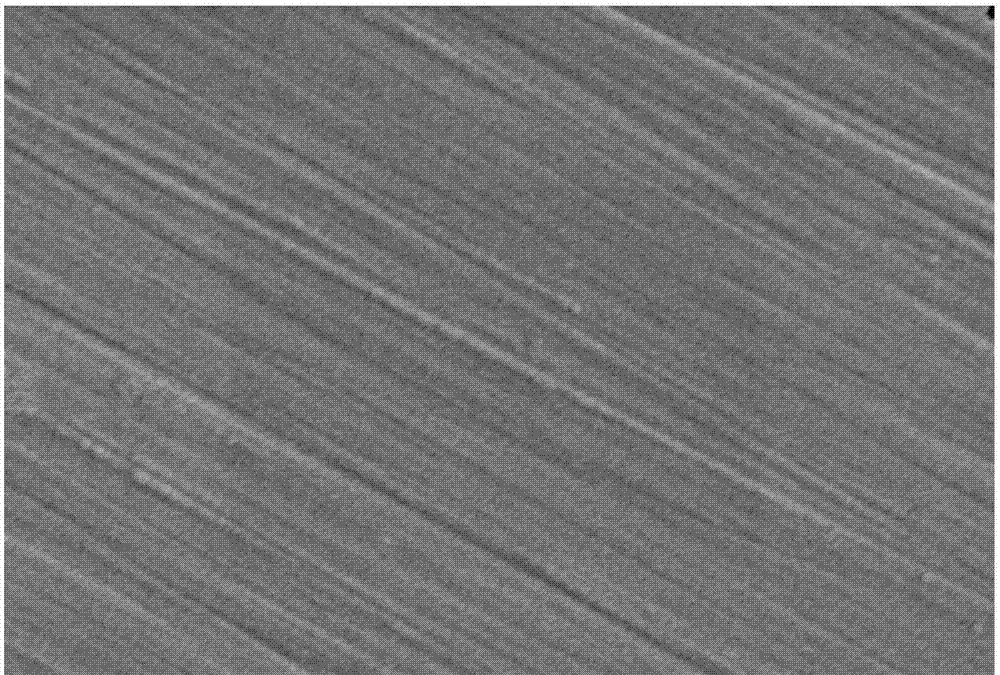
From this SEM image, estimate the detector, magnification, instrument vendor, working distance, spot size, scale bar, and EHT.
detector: SEI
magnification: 1.3 K X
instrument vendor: JEOL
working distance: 13 mm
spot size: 86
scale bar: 10000 nm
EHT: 20 kV